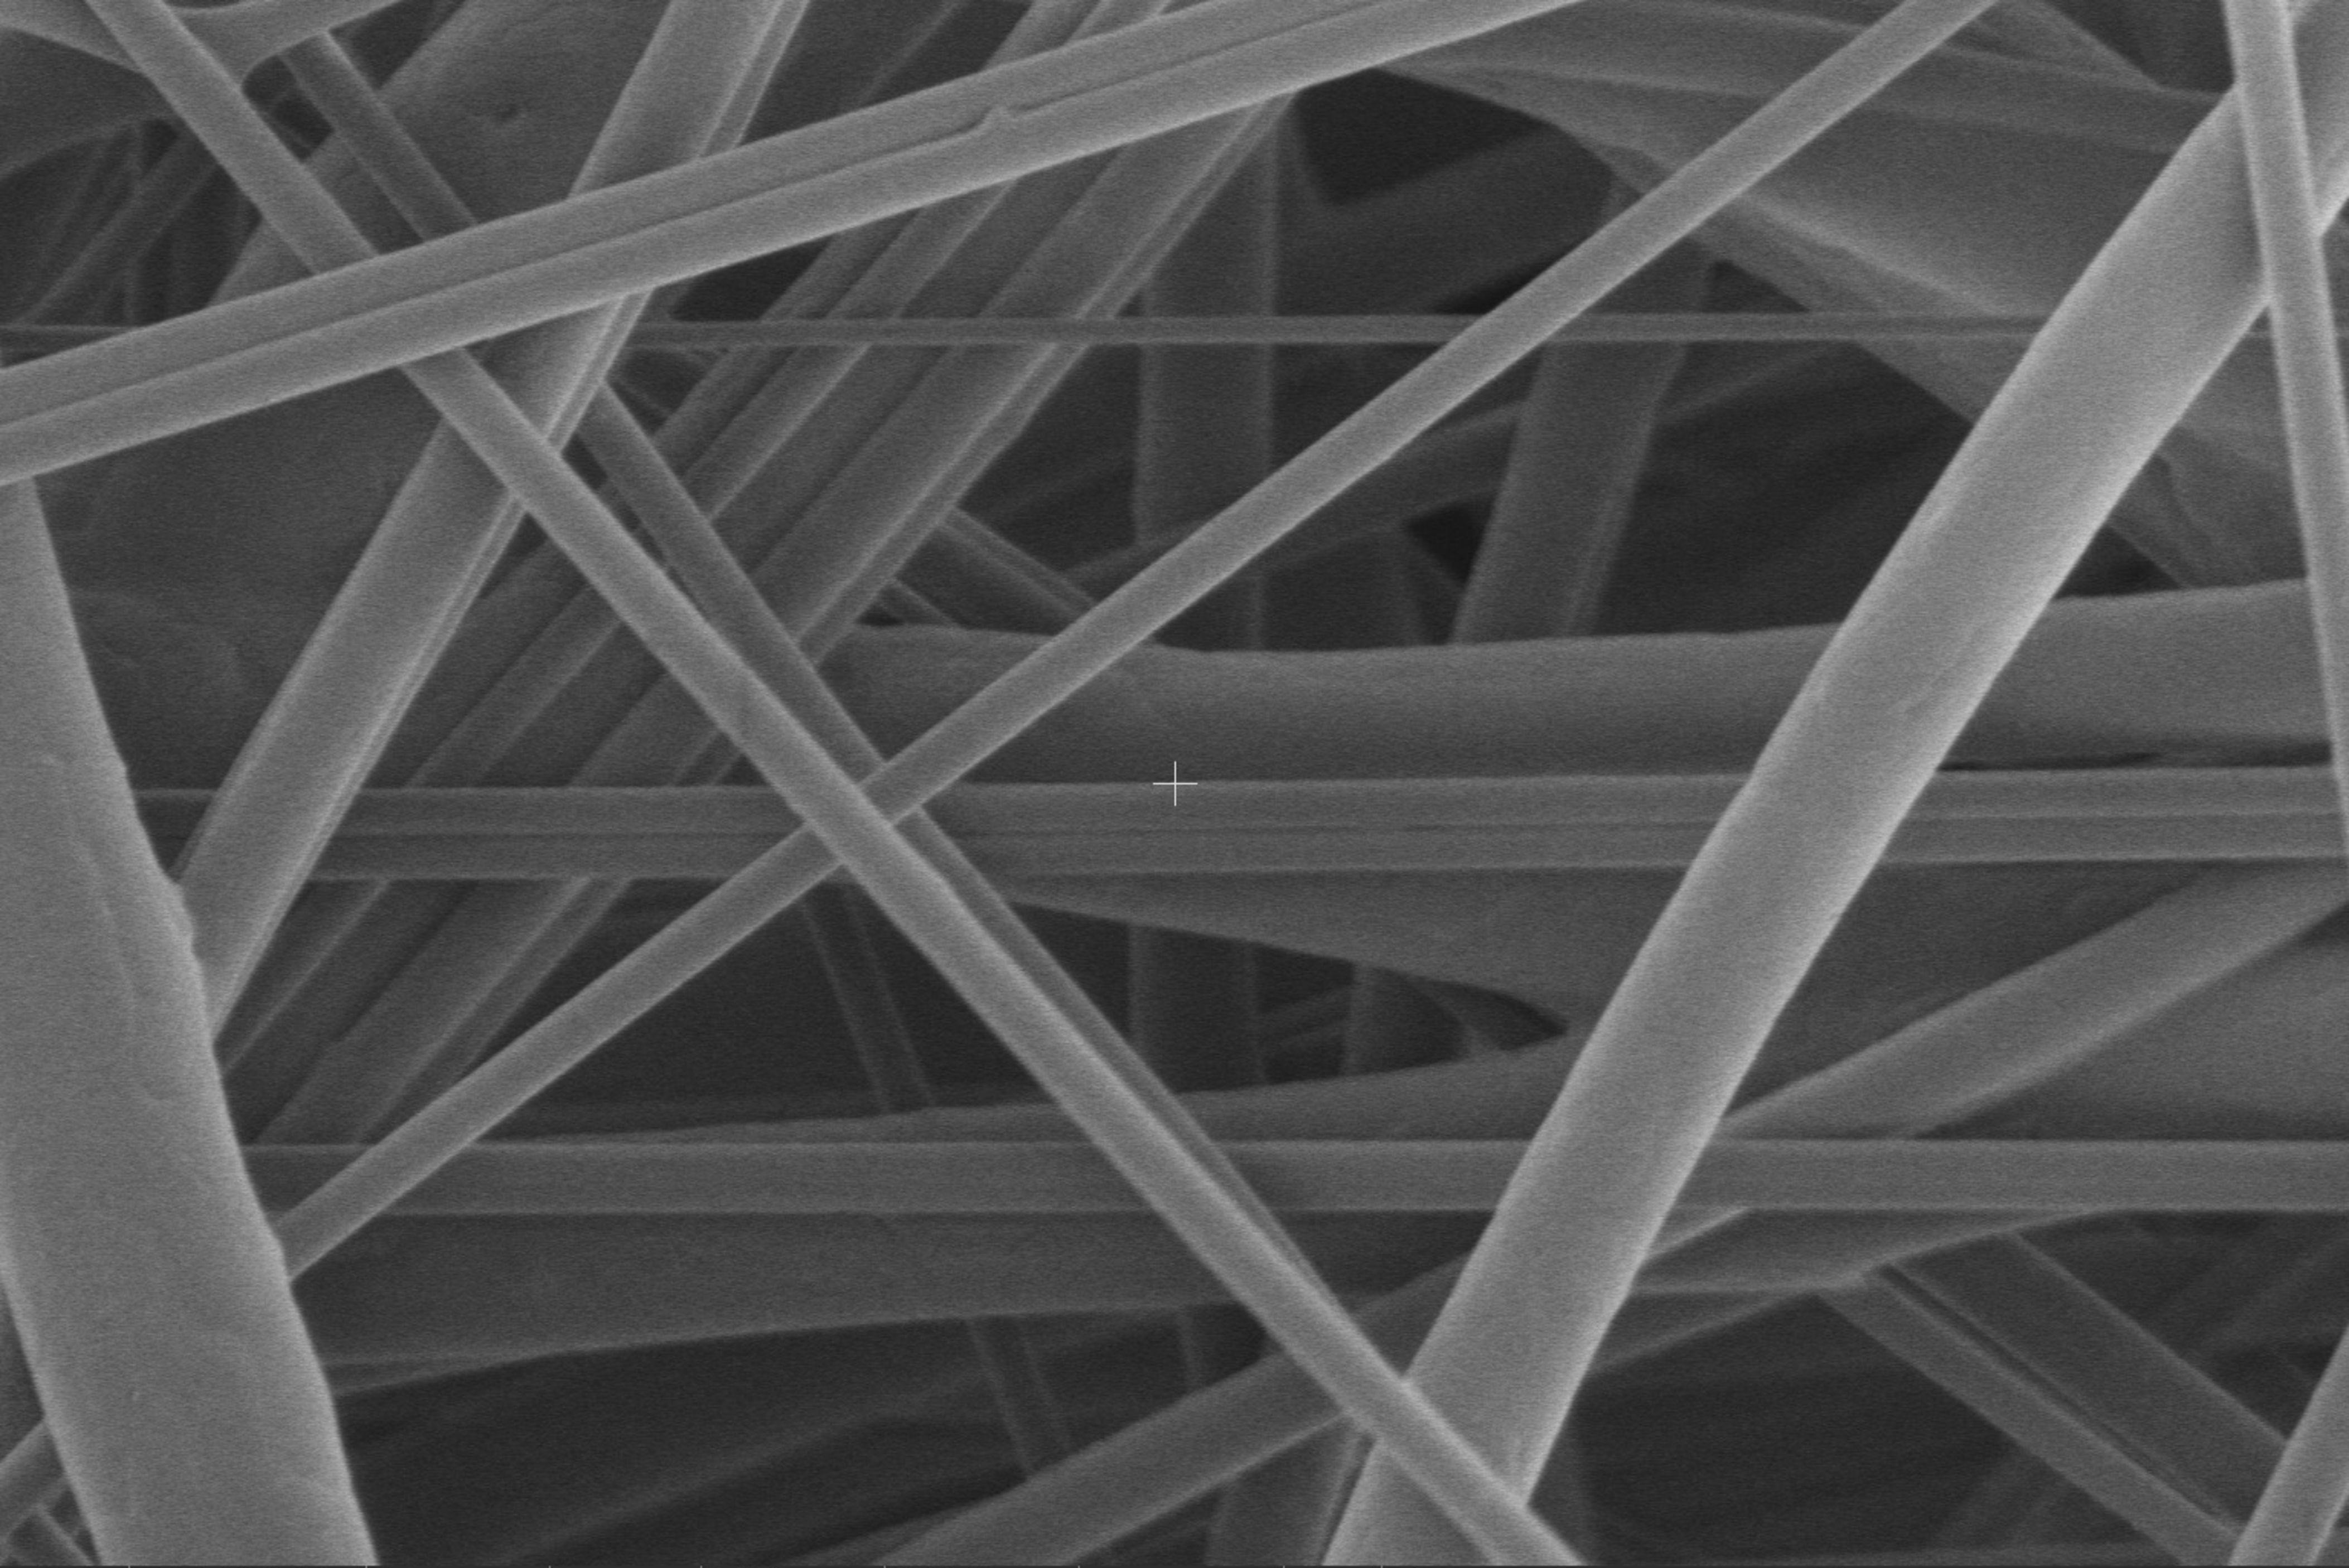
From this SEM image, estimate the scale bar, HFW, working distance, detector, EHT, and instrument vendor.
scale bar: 20000 nm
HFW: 51.8 µm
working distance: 7.4 mm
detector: T2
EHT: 1 kV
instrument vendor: Thermo